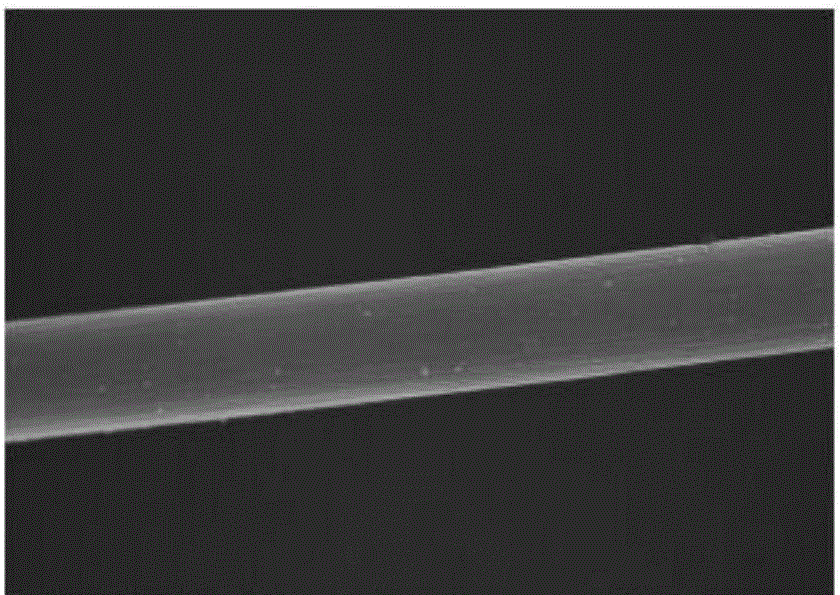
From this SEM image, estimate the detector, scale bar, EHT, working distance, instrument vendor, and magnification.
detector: TLD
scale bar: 20000 nm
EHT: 15 kV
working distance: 5.6 mm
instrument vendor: FEI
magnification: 8 K X